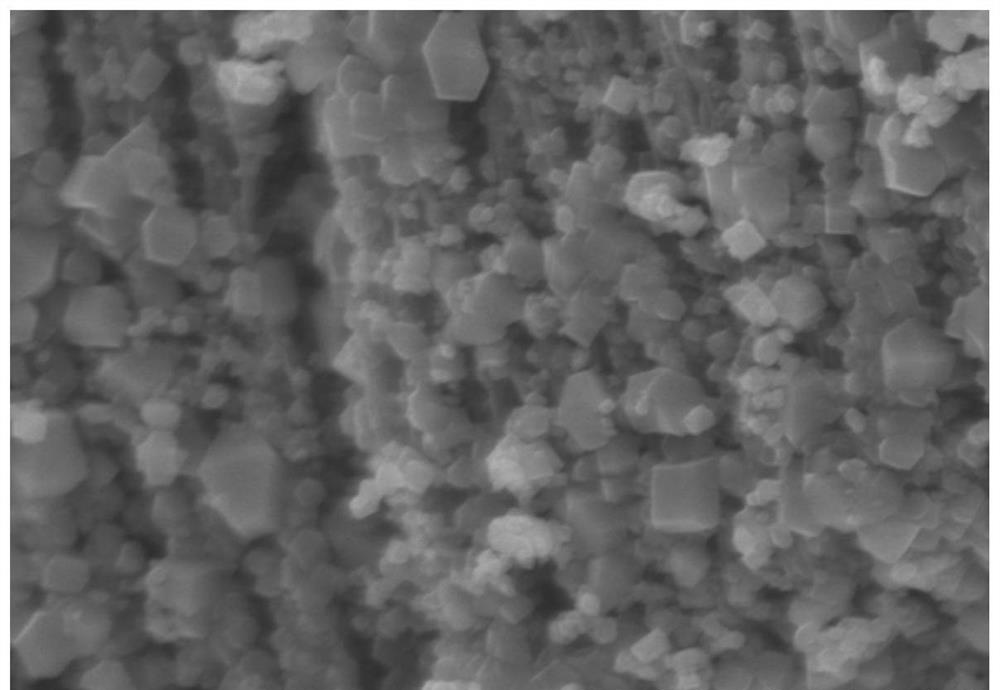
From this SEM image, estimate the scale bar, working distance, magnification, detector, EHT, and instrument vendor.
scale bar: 1000 nm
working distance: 7.8 mm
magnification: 50 K X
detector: SE(M)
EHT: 5 kV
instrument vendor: Hitachi